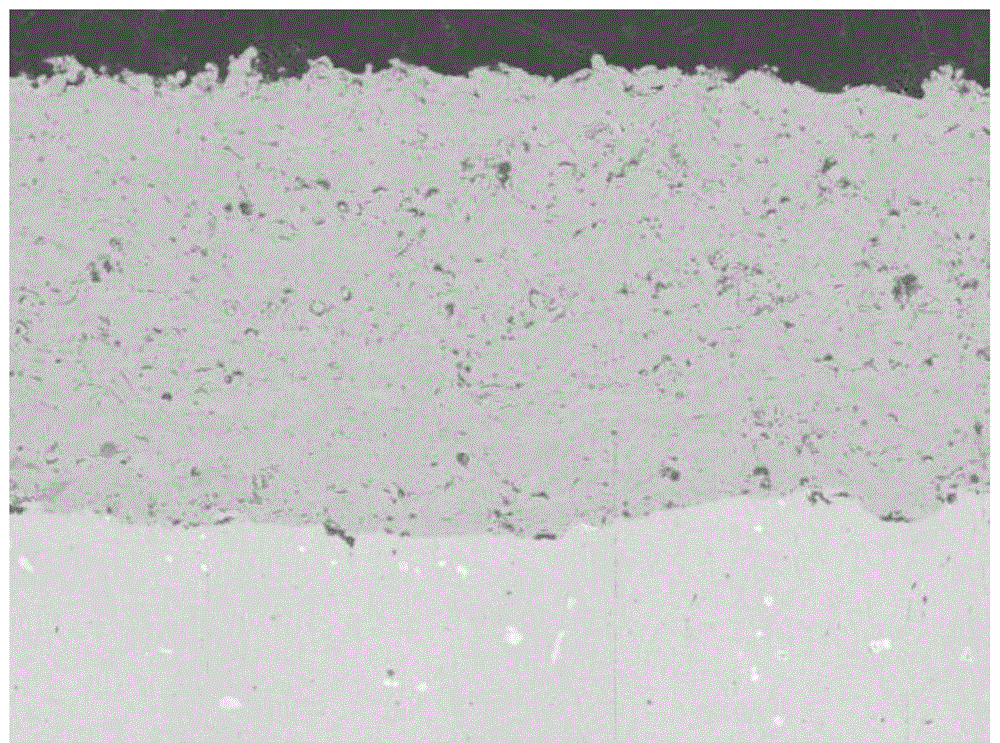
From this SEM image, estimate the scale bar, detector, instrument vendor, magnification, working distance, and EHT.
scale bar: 100000 nm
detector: BSE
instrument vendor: Tescan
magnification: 0.5 K X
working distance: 12.9 mm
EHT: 15 kV